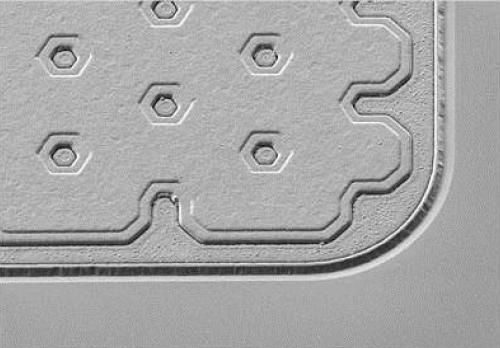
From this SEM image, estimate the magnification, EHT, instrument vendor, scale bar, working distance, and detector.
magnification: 1.64 K X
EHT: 3 kV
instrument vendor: Zeiss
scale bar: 10000 nm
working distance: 4.9 mm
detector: SE2